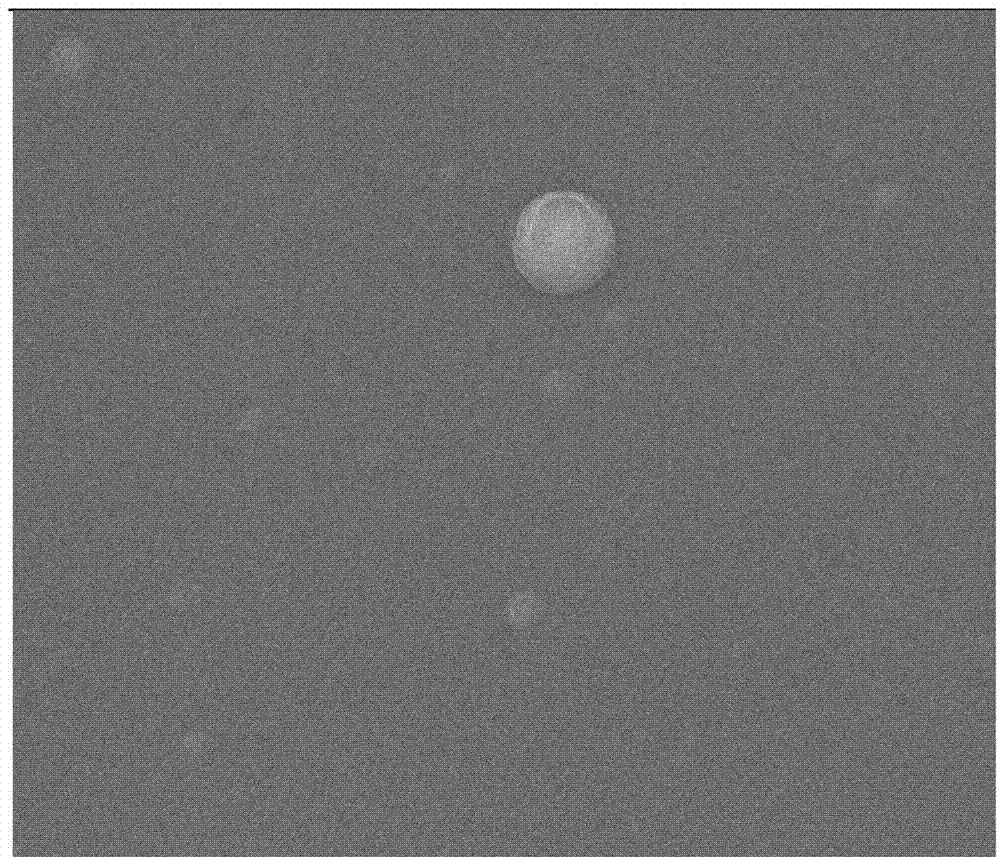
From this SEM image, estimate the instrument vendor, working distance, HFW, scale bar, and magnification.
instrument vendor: FEI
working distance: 8.7 mm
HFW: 27.04 µm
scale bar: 10000 nm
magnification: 5 K X